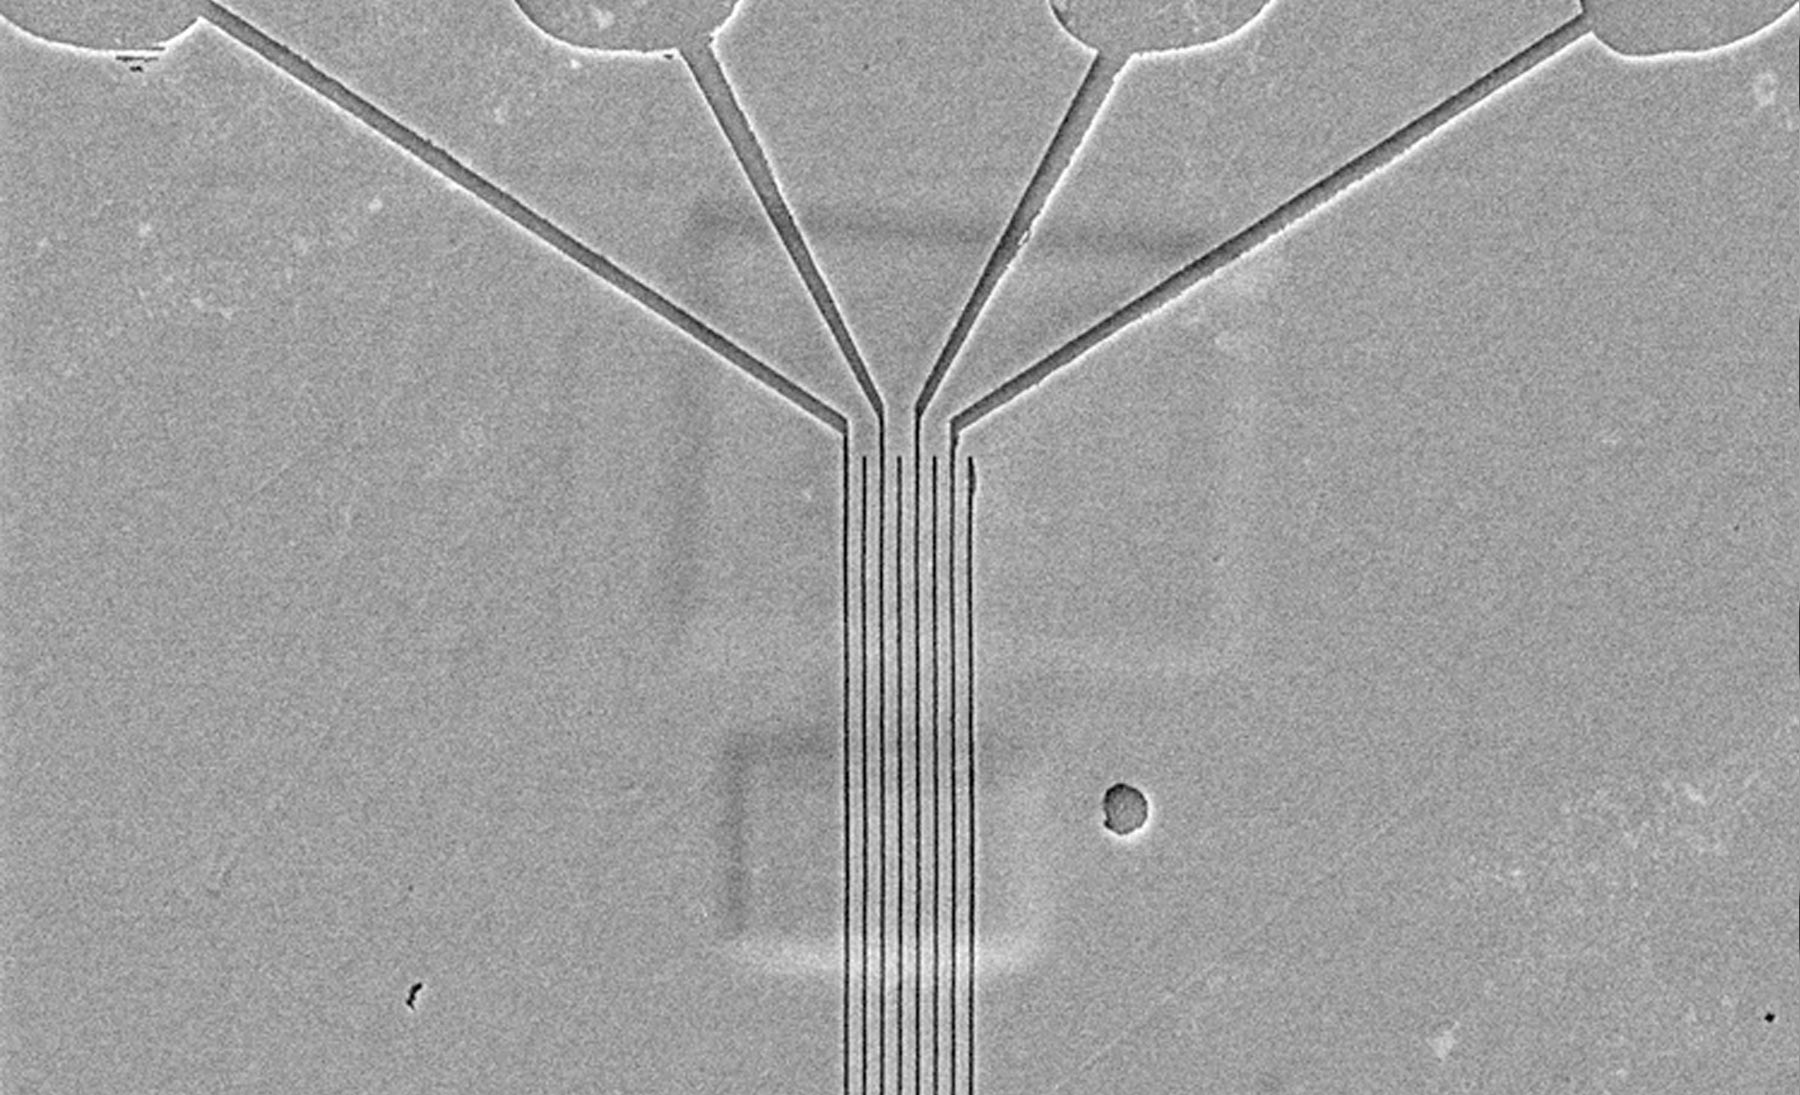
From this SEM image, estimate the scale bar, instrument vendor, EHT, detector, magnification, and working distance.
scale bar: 1000 nm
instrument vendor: JEOL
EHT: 3 kV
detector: LEI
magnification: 4.5 K X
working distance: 11 mm